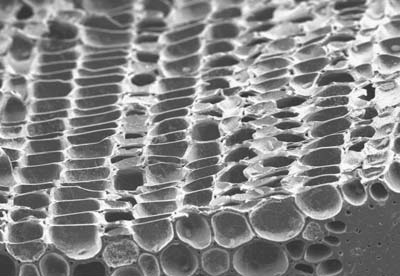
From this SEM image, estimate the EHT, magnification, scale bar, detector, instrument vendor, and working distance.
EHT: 5 kV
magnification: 0.27 K X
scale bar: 100000 nm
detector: SEI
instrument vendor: JEOL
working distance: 10 mm